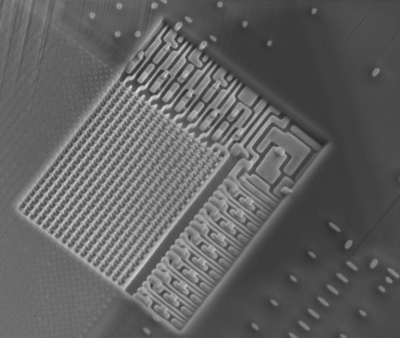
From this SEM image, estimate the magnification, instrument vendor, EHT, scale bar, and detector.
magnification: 24 K X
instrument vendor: FEI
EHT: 5 kV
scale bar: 5000 nm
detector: ETD